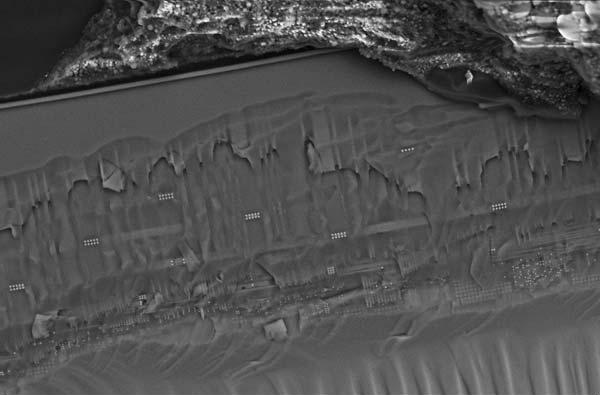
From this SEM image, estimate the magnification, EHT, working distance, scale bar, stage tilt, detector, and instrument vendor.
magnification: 1.82 K X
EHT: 15 kV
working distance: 2.5 mm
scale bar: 10000 nm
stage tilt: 46.8°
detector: InLens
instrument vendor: Zeiss